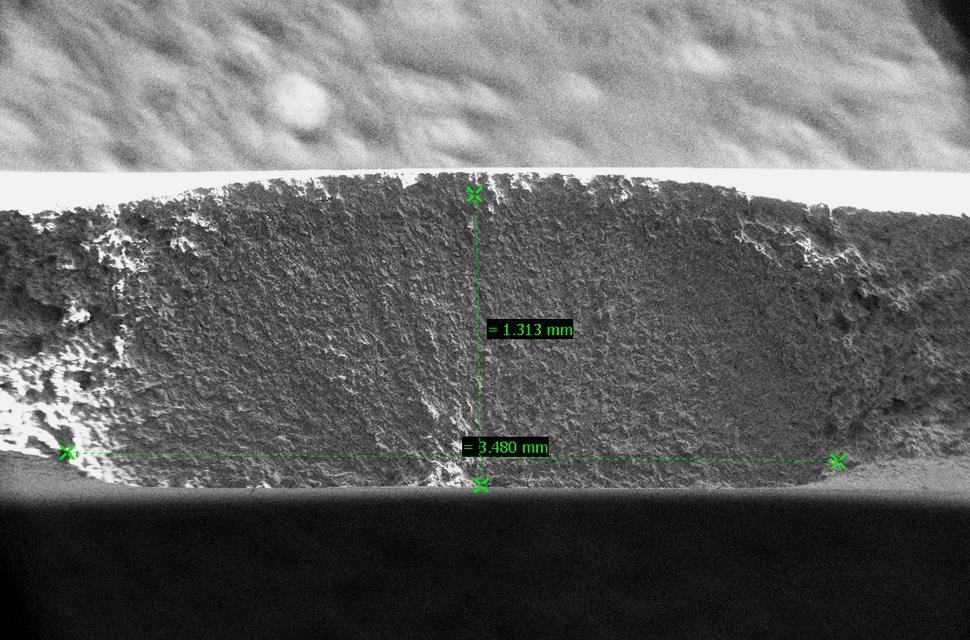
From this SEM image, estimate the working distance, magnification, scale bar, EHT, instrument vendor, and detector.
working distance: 9 mm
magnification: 0.061 K X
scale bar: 300000 nm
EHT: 20 kV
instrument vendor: Zeiss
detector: SE1